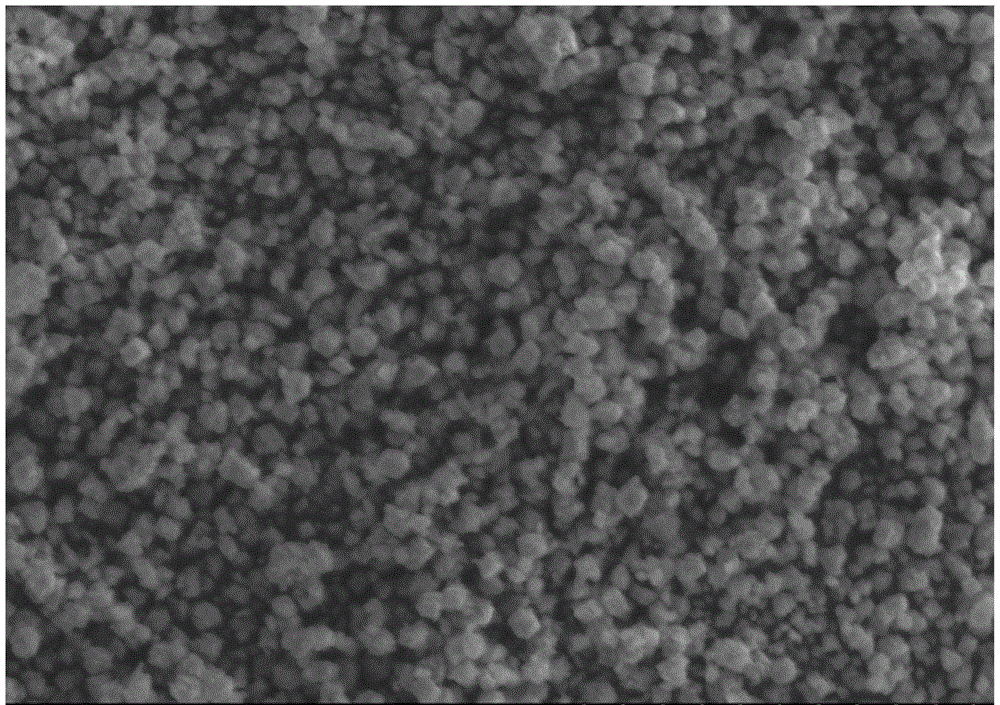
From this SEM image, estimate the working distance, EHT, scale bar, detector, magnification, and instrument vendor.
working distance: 10.8 mm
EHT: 15 kV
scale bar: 50000 nm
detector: SE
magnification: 1 K X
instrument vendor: Hitachi